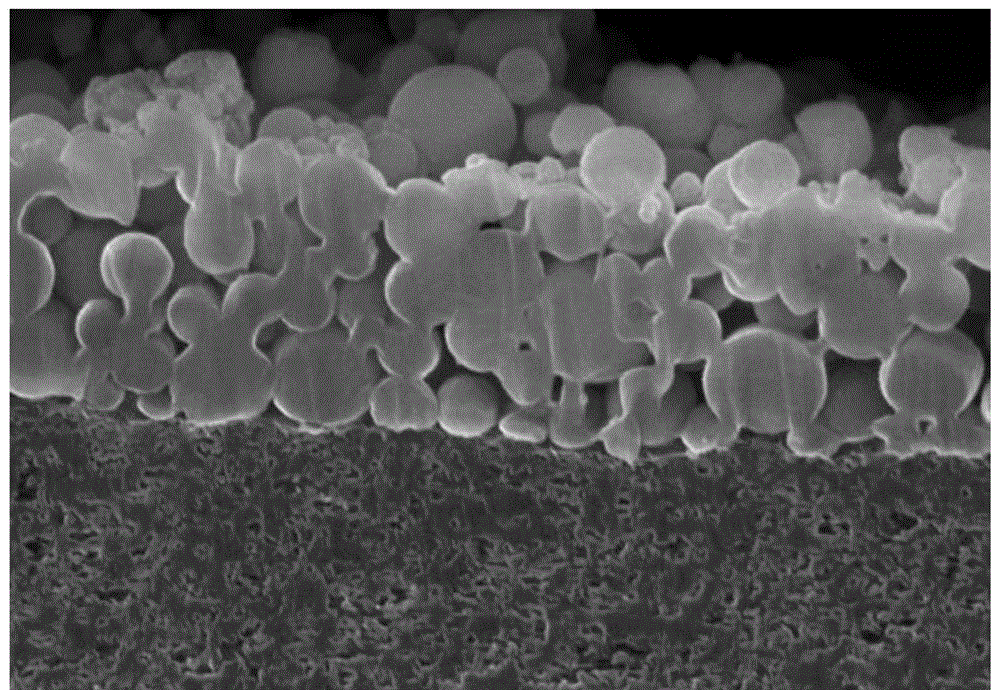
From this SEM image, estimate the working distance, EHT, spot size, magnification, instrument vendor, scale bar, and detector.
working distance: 6.1 mm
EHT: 10 kV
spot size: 4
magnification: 50 K X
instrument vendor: FEI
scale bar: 3000 nm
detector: TLD